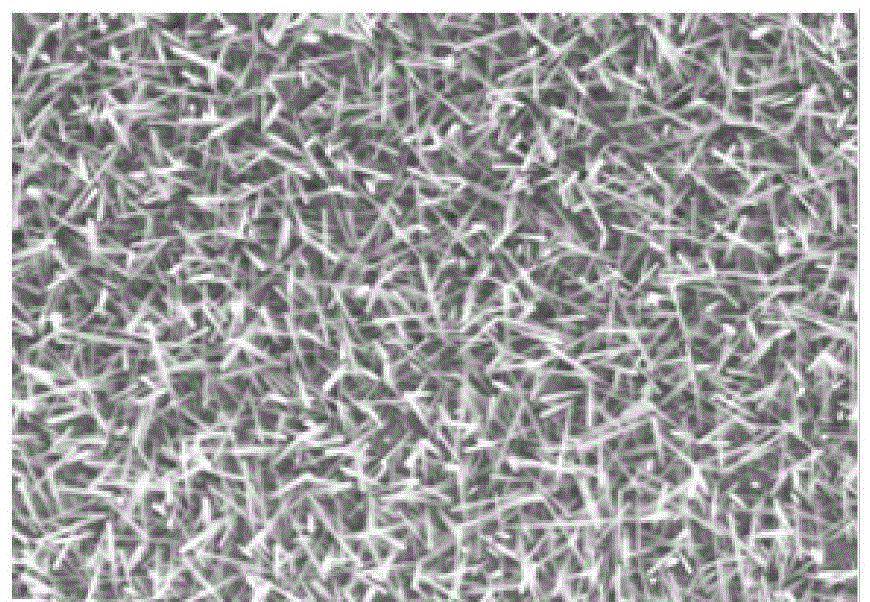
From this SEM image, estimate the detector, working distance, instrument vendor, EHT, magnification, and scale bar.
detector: SE(M)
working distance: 6.9 mm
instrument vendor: Hitachi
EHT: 5 kV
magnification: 50 K X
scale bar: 1000 nm